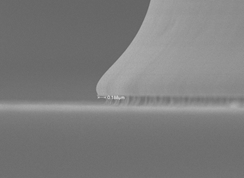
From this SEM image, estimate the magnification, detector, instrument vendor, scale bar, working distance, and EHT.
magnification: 20 K X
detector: SEI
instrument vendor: JEOL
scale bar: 1000 nm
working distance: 8 mm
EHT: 10 kV